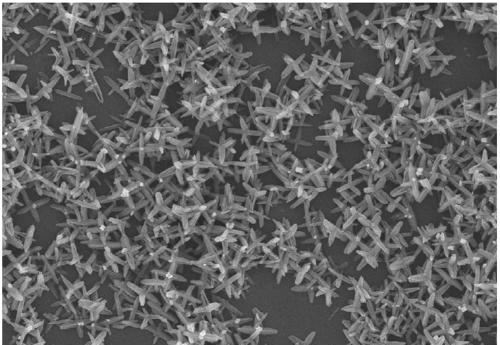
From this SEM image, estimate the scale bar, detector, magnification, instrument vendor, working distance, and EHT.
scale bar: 5000 nm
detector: SE(UL)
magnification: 10 K X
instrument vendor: Hitachi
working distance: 8.5 mm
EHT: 5 kV